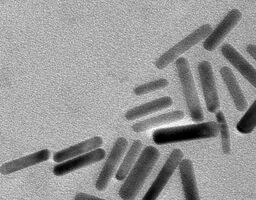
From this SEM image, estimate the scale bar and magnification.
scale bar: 100 nm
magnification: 97 K X